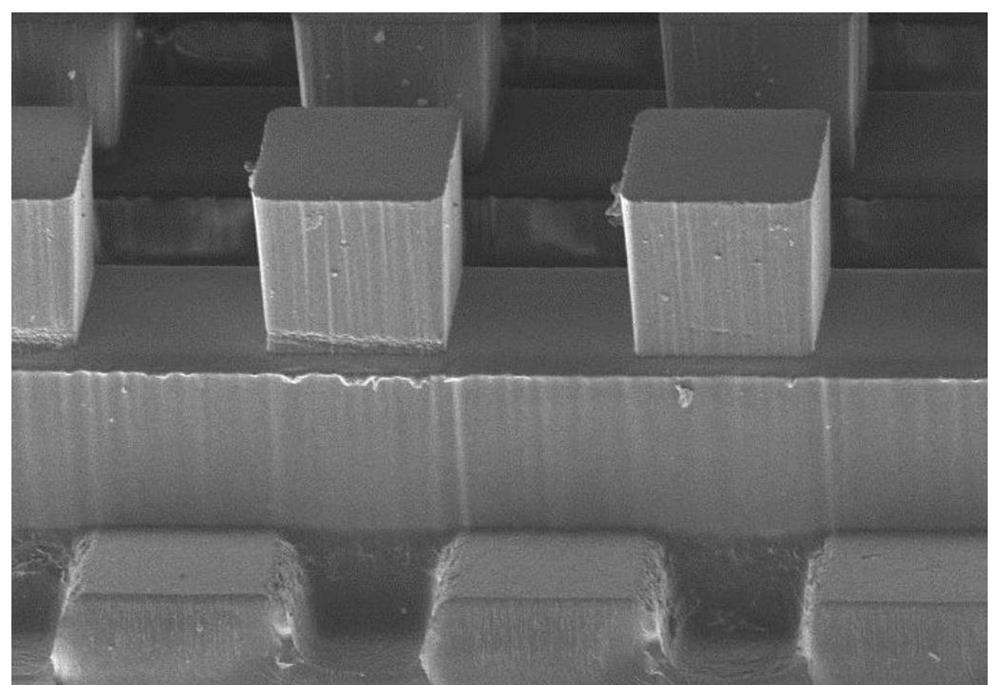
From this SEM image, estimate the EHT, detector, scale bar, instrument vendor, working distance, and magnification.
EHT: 3 kV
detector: LM(L)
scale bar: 400000 nm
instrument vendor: Hitachi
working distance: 9.1 mm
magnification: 0.13 K X